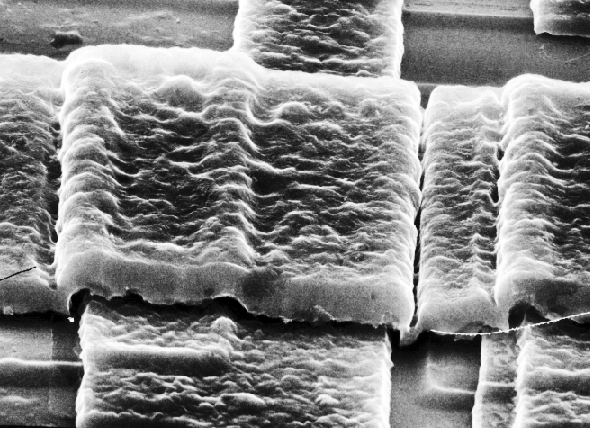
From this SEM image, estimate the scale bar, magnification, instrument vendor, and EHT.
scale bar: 1810 nm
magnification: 5.51 K X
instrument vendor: JEOL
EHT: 20 kV